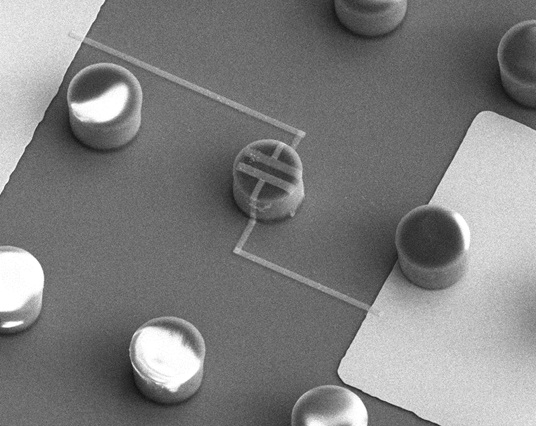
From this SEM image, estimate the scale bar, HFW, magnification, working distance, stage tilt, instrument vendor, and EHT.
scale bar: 30000 nm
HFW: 85.3 µm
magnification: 1.75 K X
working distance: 4.3 mm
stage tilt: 30°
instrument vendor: FEI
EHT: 10 kV